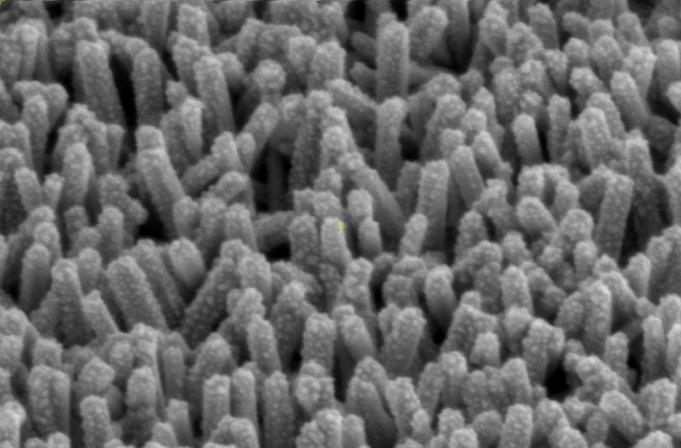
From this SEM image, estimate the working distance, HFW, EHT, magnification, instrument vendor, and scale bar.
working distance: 3.9 mm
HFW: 1.38 µm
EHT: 2 kV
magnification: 150 K X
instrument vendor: FEI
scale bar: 500 nm